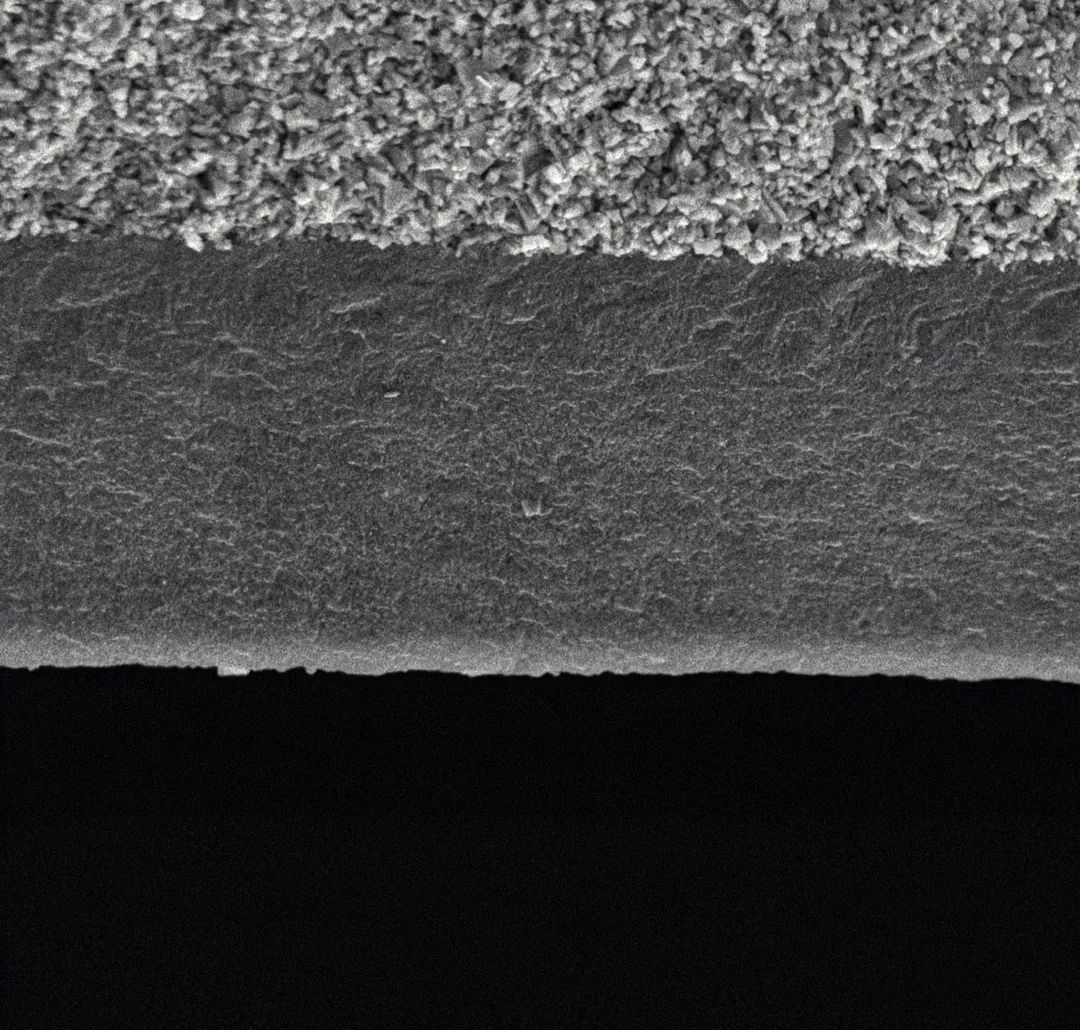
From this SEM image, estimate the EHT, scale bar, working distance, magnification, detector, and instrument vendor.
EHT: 15 kV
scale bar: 5000 nm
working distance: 4.9 mm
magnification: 10 K X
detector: SE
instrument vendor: other